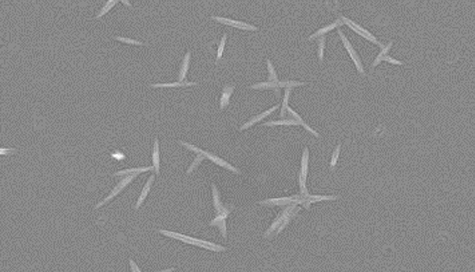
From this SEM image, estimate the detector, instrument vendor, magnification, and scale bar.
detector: SE(M)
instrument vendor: Hitachi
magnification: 20 K X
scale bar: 2000 nm